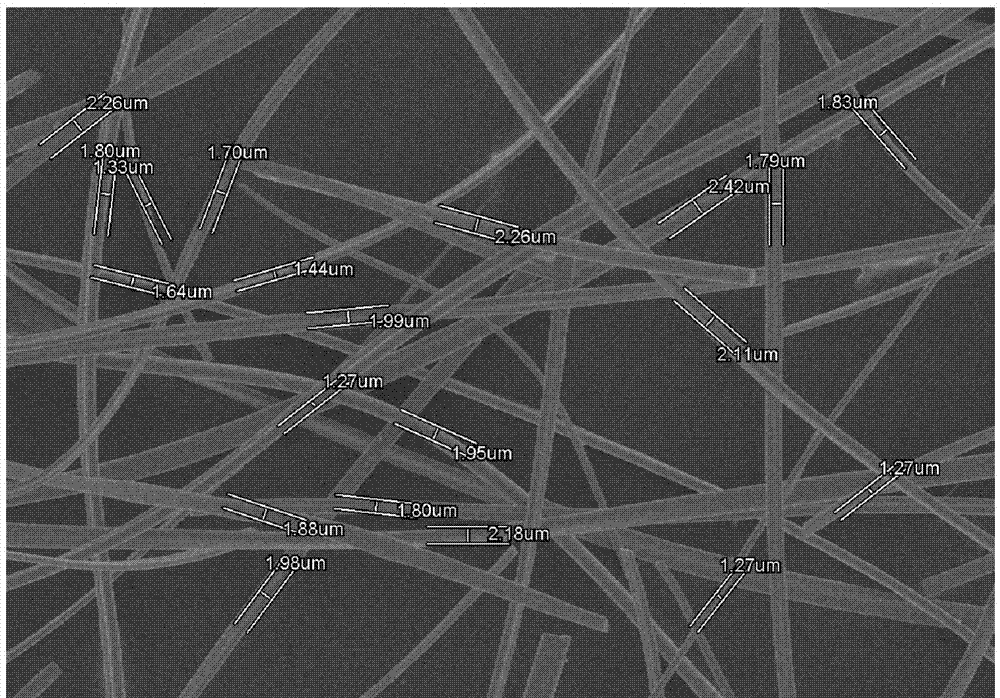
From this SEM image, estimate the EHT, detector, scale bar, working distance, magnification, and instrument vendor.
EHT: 5 kV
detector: SE(M)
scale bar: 50000 nm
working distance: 7.4 mm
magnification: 1 K X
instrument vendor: Hitachi